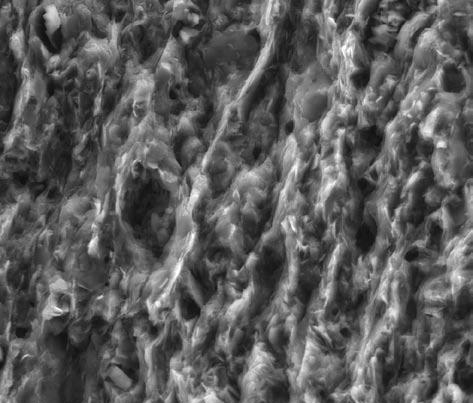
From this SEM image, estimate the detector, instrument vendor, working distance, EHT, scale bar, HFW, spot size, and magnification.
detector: LVD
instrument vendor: FEI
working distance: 5 mm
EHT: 18 kV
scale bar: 30000 nm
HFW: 74.6 µm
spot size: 4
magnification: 4 K X